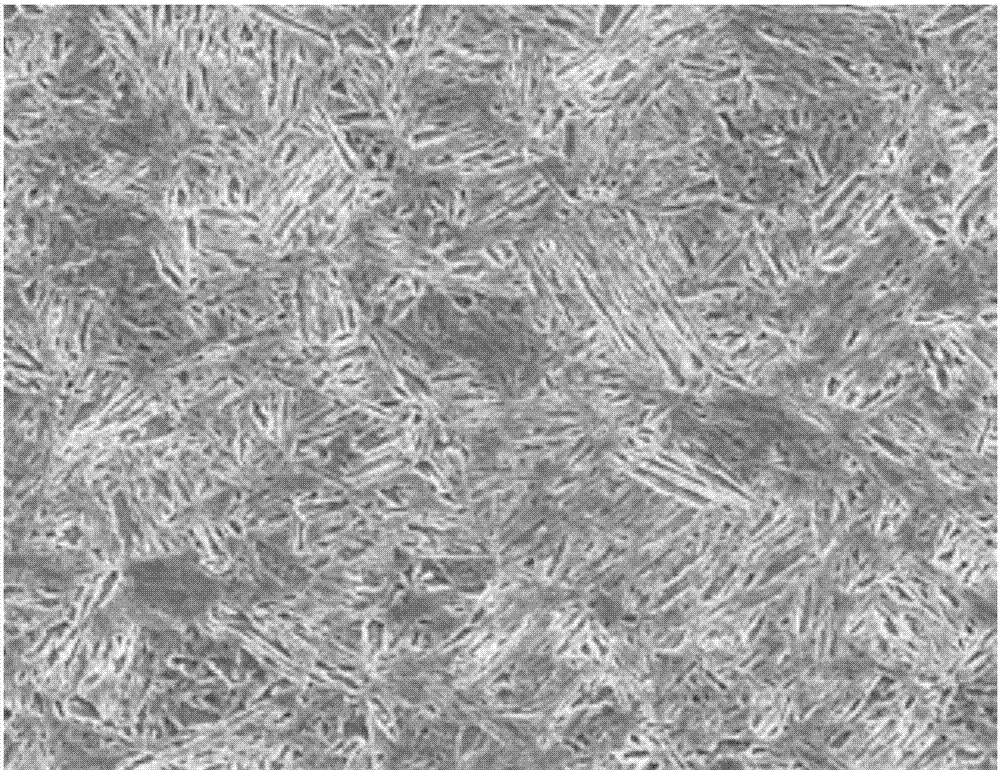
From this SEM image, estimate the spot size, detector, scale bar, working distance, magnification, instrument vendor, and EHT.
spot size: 4.5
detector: ETD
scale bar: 50000 nm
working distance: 9.6 mm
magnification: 1 K X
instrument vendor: FEI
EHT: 20 kV